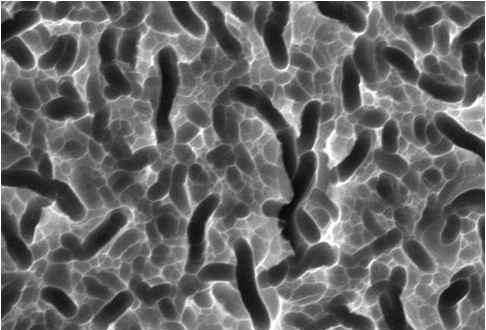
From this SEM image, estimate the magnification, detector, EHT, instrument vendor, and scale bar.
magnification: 3 K X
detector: SEI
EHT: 20 kV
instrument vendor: JEOL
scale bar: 5000 nm